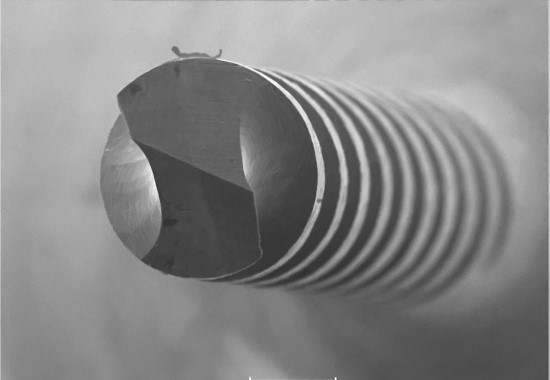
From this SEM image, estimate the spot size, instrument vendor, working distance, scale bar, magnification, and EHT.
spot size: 8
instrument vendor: JEOL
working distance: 20 mm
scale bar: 30000 nm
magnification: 1 K X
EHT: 20 kV